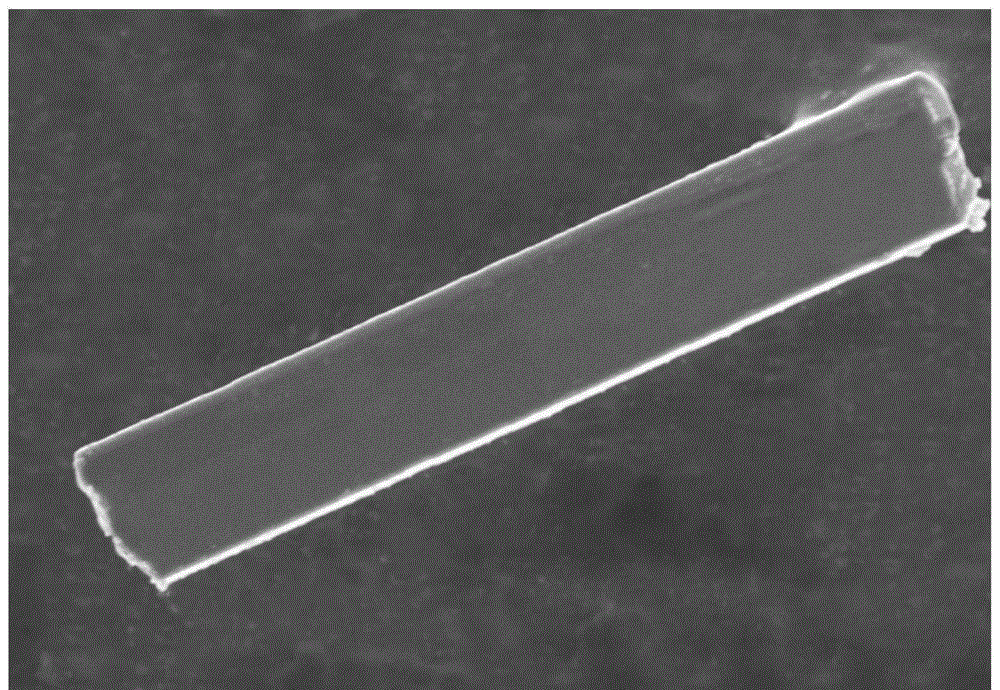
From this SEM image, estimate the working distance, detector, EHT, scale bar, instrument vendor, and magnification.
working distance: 8.2 mm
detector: SE(UL)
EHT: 5 kV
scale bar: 10000 nm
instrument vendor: Hitachi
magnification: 3.5 K X